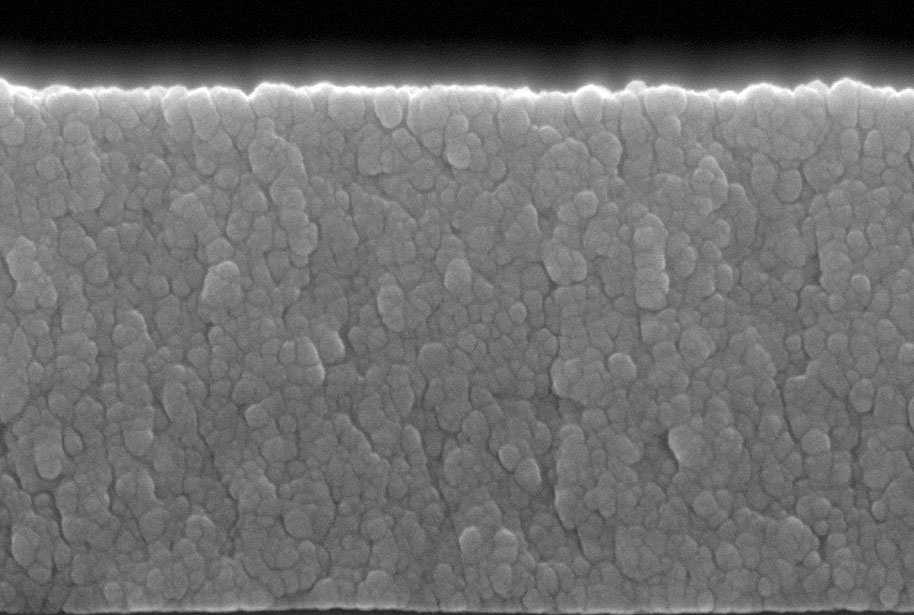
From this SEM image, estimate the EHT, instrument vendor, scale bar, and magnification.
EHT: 10 kV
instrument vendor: JEOL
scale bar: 100 nm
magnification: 100 K X